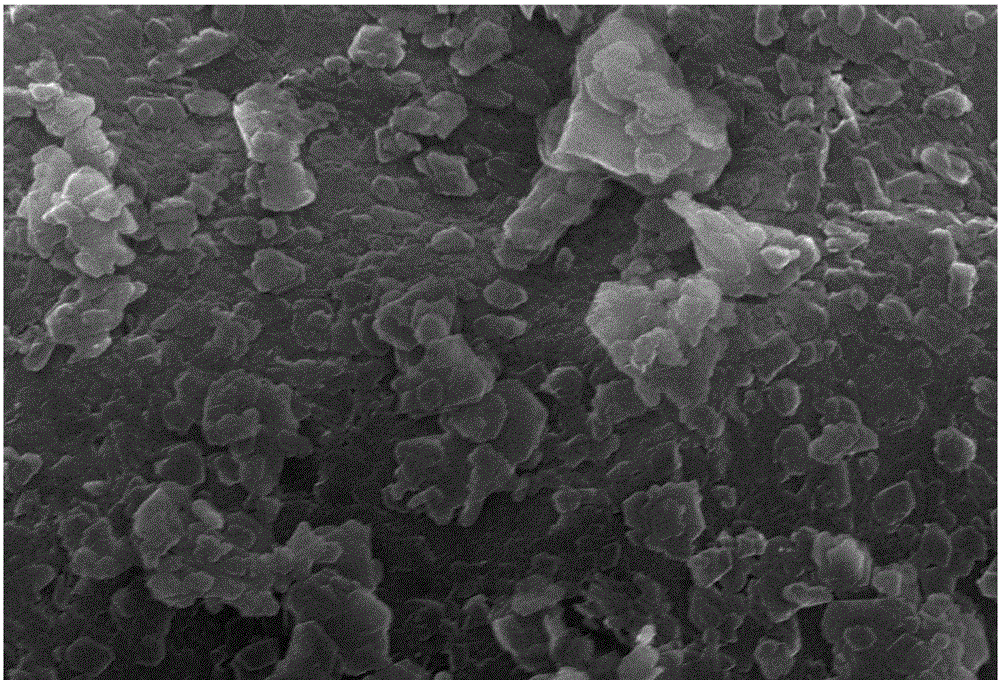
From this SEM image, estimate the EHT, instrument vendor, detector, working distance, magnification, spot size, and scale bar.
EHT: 30 kV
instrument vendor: JEOL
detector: SEI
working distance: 11 mm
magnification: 10 K X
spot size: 30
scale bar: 1000 nm